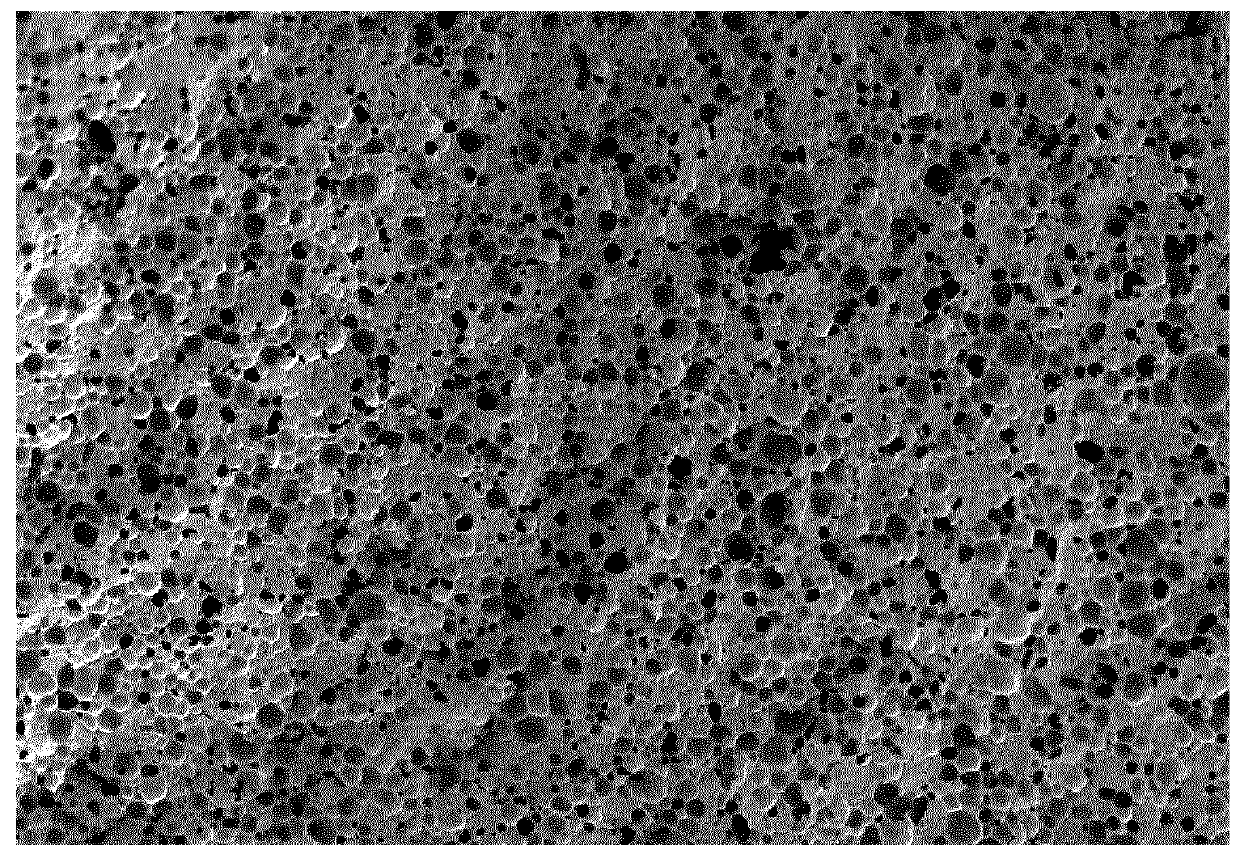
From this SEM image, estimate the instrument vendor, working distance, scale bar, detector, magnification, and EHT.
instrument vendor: Hitachi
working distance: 8.9 mm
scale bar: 1000000 nm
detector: SE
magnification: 0.05 K X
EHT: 3 kV